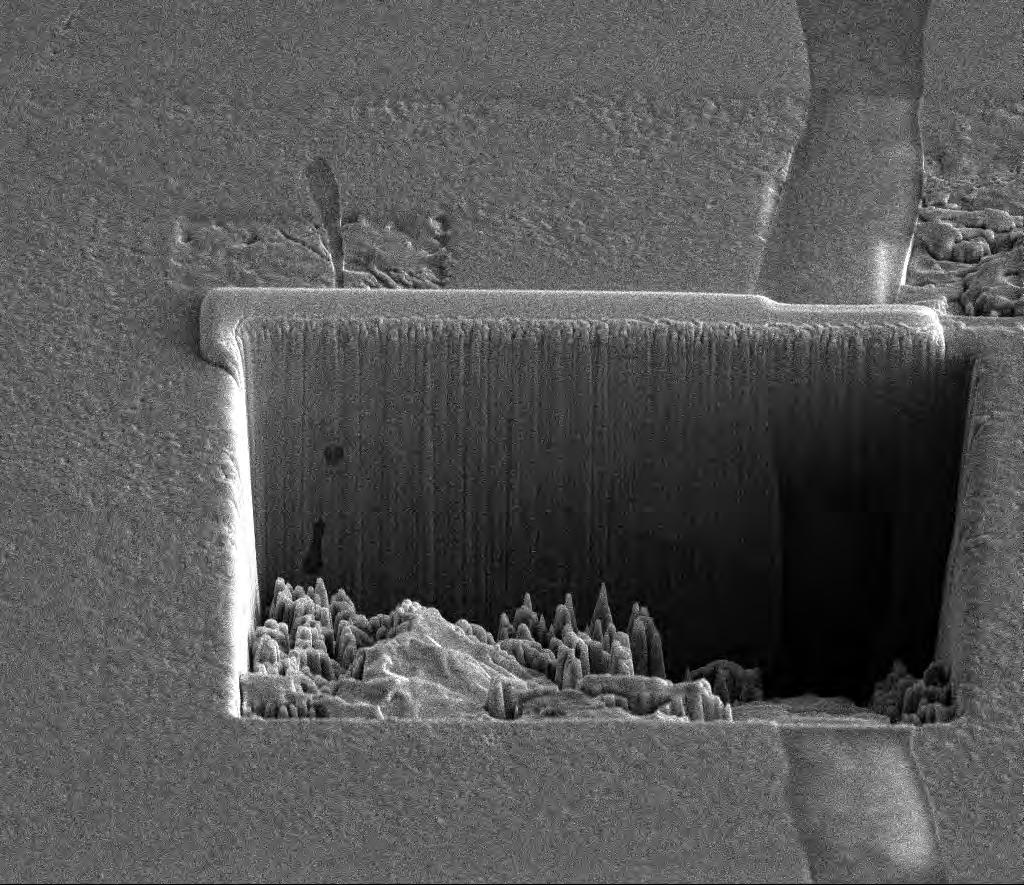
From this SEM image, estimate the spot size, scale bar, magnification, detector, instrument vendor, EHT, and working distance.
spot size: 4.5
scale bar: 20000 nm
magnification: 3.5 K X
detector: ETD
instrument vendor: FEI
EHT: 10 kV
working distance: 10 mm